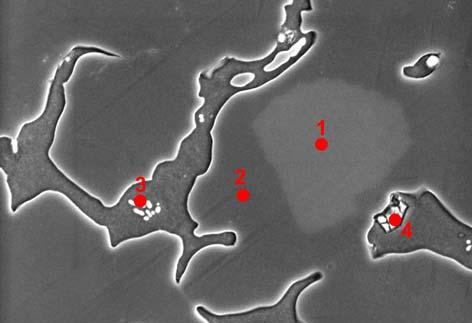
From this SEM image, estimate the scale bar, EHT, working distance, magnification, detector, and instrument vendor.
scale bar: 50000 nm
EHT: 15 kV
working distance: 16 mm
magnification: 1 K X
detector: SE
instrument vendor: Hitachi